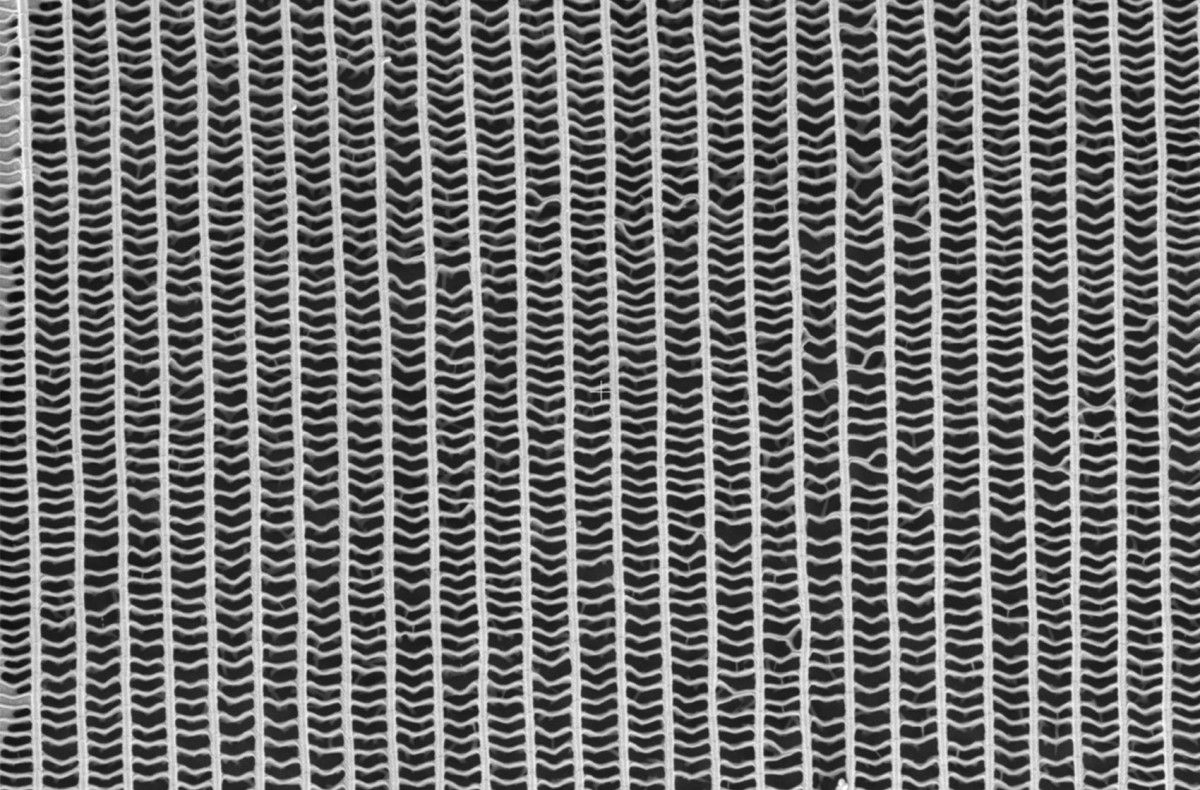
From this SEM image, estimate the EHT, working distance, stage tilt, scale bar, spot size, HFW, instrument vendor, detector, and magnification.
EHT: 5 kV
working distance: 7.1 mm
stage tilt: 0°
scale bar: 10000 nm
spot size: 10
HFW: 41.4 µm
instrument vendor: FEI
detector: ETD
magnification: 10 K X